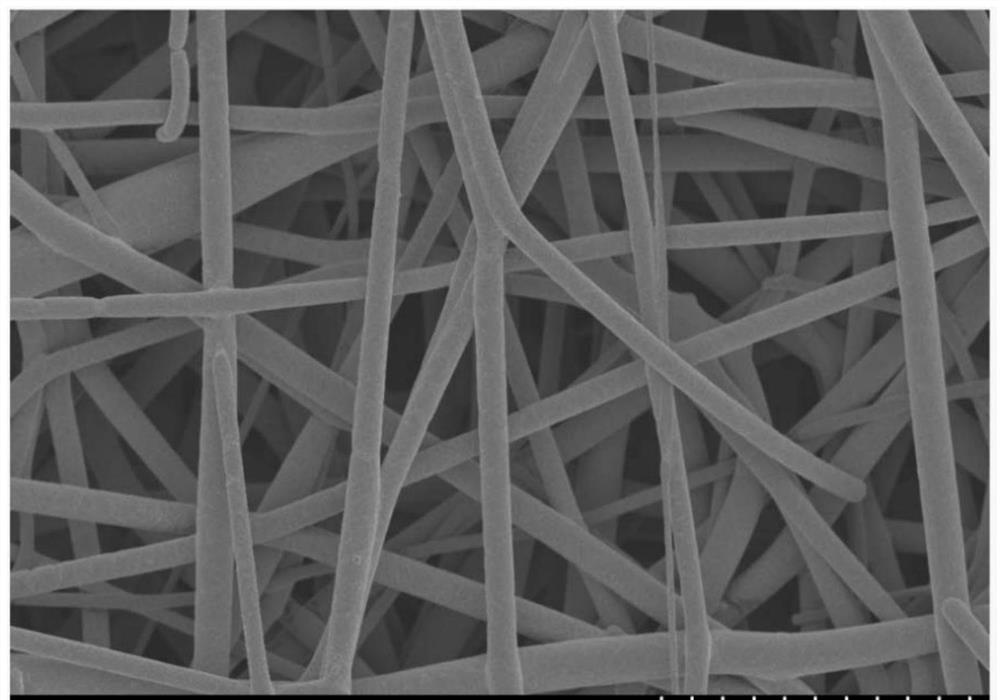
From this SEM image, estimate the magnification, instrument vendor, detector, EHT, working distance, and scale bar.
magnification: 2 K X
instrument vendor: Hitachi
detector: SE(M)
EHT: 3 kV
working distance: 8.7 mm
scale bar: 20000 nm